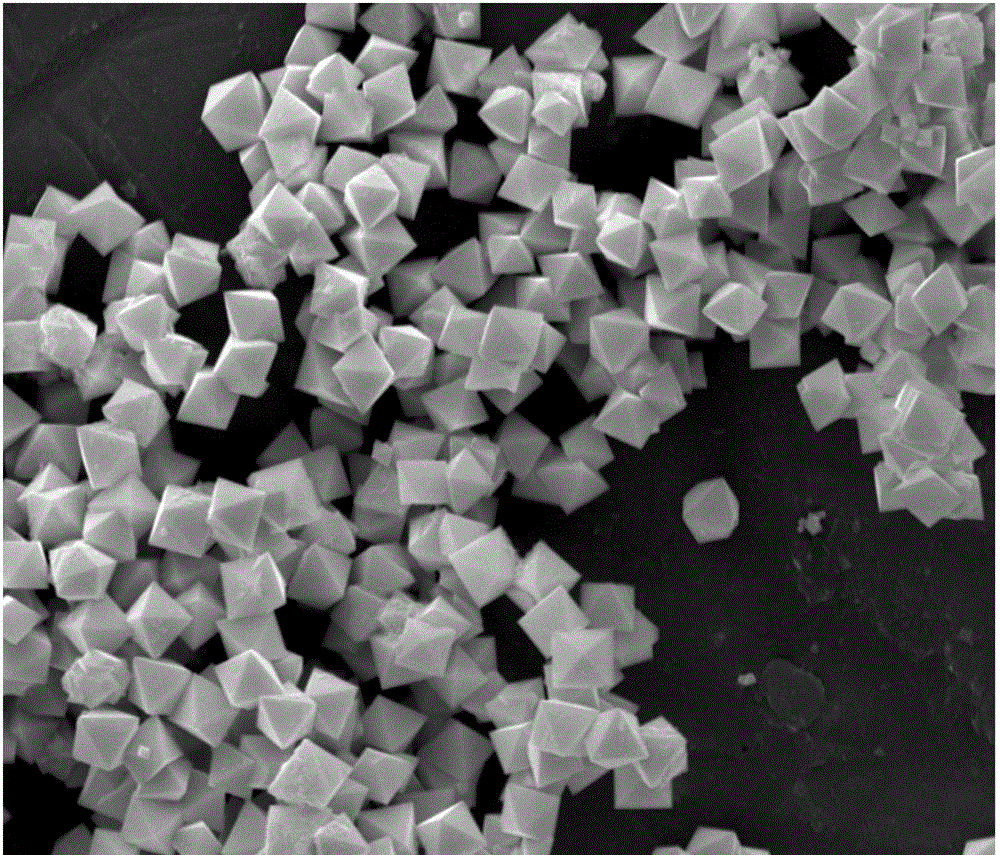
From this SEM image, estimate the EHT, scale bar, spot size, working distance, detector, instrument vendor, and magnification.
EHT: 20 kV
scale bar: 5000 nm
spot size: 3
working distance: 7.6 mm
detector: ETD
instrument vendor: FEI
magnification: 12 K X